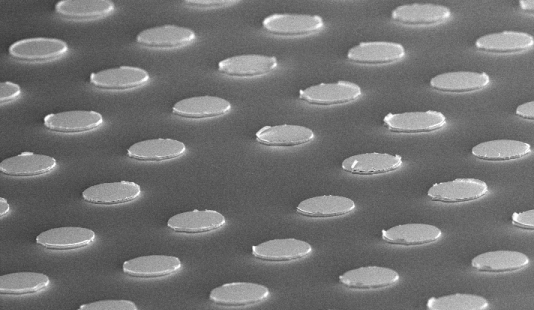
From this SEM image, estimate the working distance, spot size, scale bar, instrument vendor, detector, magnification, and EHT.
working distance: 6.3 mm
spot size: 3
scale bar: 20000 nm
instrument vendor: FEI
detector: TLD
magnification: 0.829 K X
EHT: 5 kV